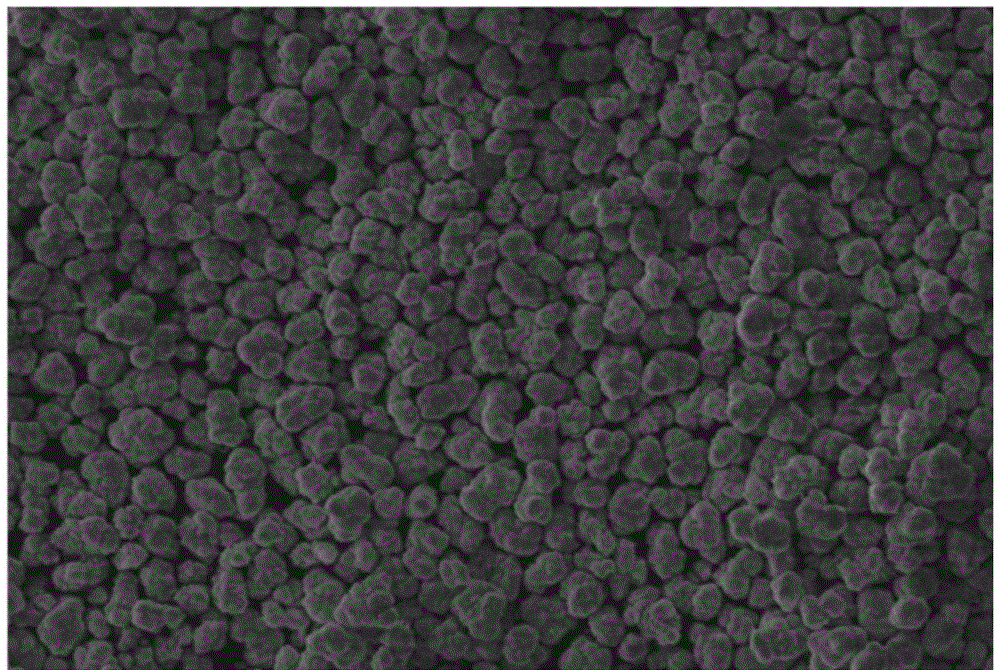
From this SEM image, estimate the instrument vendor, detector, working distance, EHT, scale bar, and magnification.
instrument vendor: Zeiss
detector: SE1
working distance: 11.5 mm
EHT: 10 kV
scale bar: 2000 nm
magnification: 5 K X